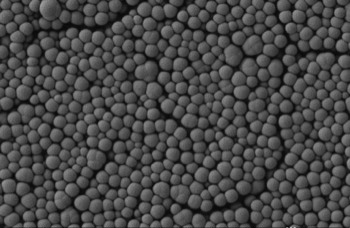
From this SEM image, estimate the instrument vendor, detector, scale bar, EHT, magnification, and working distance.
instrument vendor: Zeiss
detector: SE2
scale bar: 200 nm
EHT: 1 kV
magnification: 20 K X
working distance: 5.1 mm